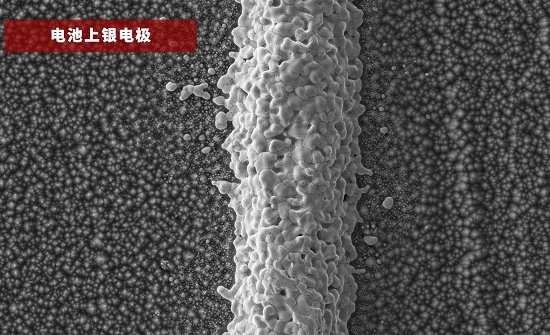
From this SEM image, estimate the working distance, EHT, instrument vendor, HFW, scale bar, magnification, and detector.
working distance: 4.829 mm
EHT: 15 kV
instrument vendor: other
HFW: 127 µm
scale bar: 30000 nm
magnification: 1 K X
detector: SED